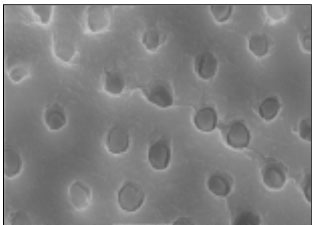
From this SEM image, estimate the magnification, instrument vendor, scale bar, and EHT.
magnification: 5 K X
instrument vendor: JEOL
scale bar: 5000 nm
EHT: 20 kV